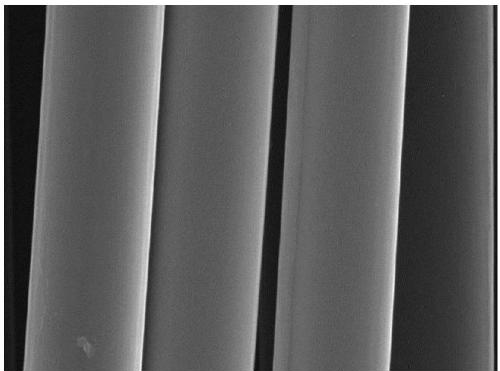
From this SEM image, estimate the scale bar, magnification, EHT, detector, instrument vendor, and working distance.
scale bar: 5000 nm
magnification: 10 K X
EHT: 10 kV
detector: SE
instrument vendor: Tescan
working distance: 15.42 mm